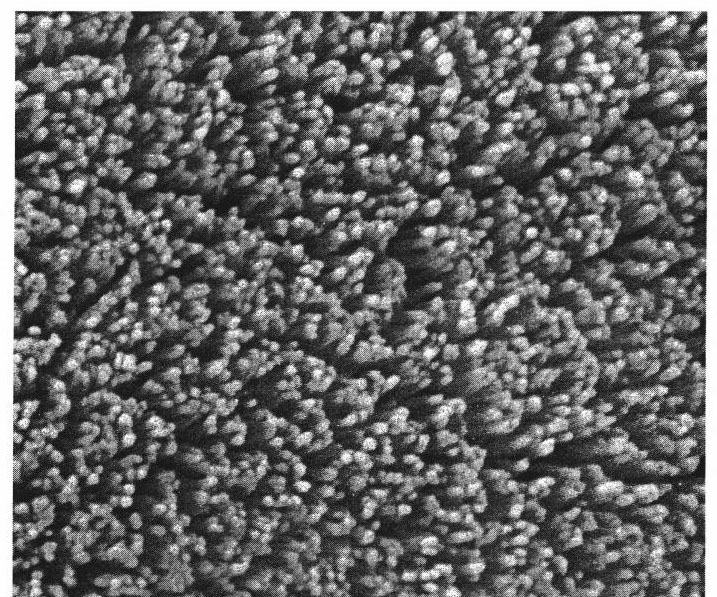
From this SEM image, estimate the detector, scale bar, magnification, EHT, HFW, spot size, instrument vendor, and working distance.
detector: SE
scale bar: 3000 nm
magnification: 20 K X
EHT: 25 kV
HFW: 7.5 µm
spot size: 3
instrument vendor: FEI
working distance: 7.4 mm